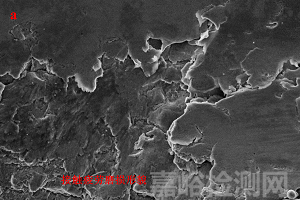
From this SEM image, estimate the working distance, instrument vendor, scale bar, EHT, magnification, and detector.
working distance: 22.1 mm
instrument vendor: Zeiss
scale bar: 10000 nm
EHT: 15 kV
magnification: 1 K X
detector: SE2